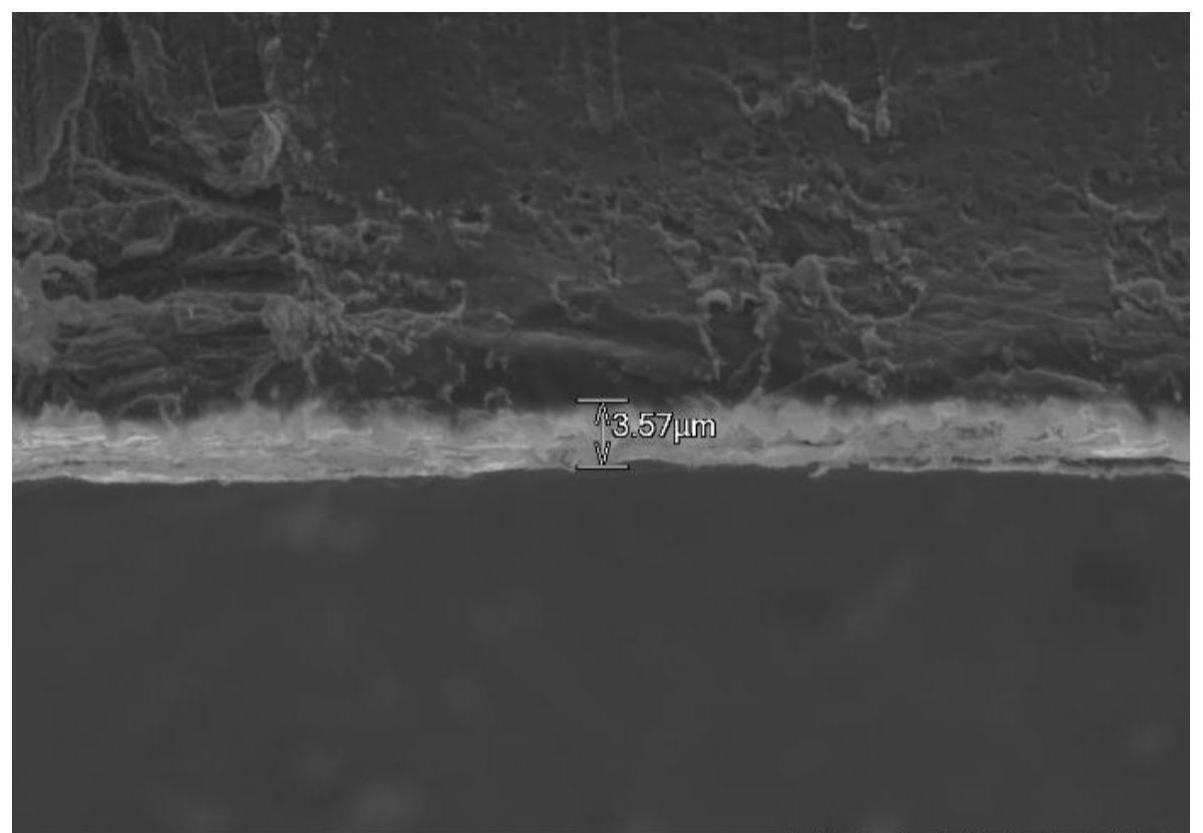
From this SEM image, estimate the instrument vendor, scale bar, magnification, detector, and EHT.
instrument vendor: Hitachi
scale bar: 20000 nm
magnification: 2 K X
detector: LM(UL)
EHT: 4 kV